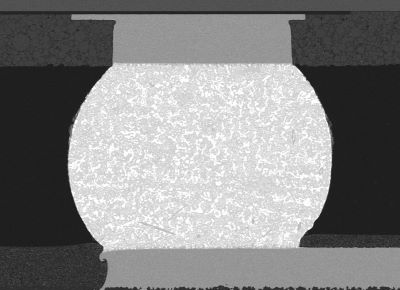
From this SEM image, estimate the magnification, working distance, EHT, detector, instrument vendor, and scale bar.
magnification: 0.3 K X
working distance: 10 mm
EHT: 10 kV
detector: BED-C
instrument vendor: JEOL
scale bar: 50000 nm